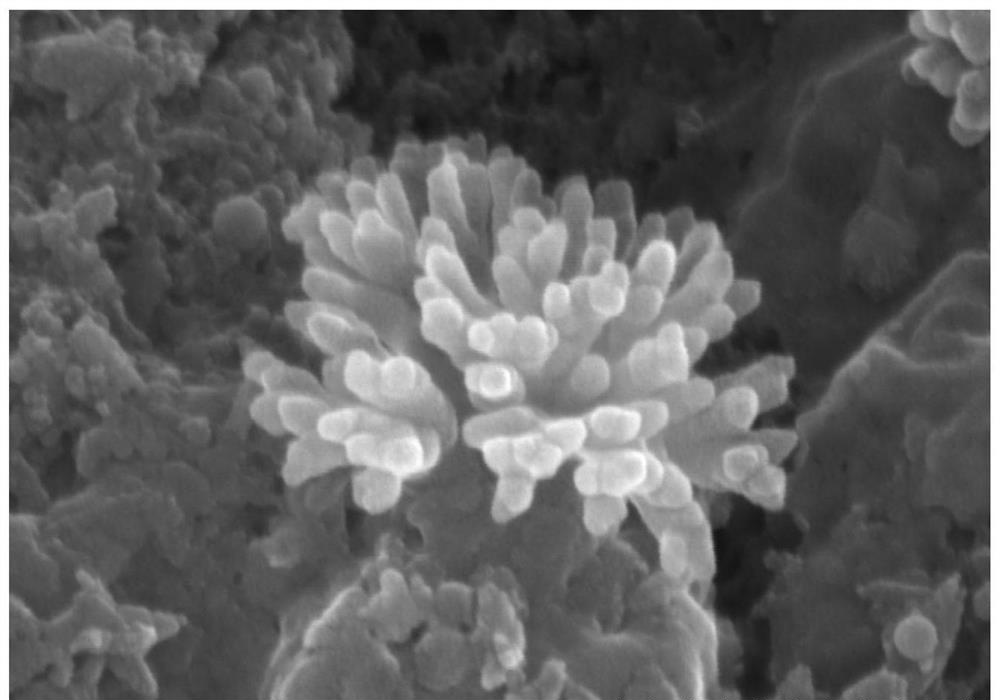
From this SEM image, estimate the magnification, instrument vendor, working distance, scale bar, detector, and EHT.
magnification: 150 K X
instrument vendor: Hitachi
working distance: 9 mm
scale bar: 300 nm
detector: SE(U)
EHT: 10 kV